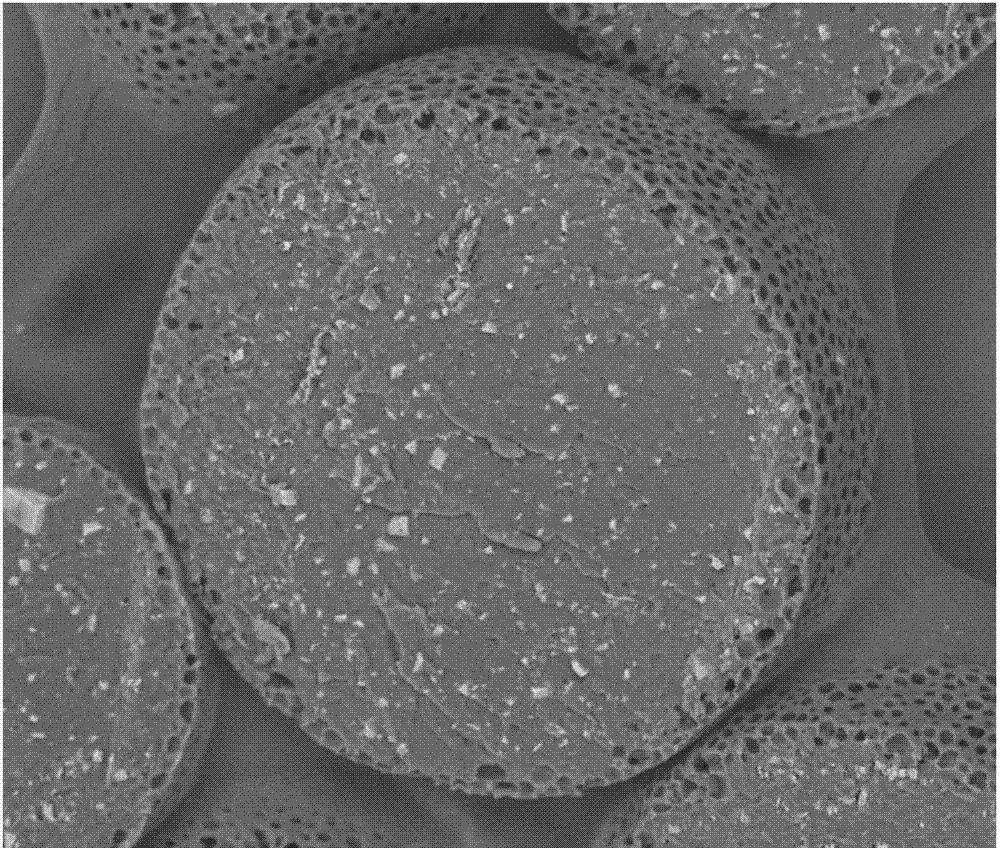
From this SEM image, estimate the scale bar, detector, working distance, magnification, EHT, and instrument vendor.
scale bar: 100000 nm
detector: DualBSD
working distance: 14 mm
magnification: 1 K X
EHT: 15 kV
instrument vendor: FEI